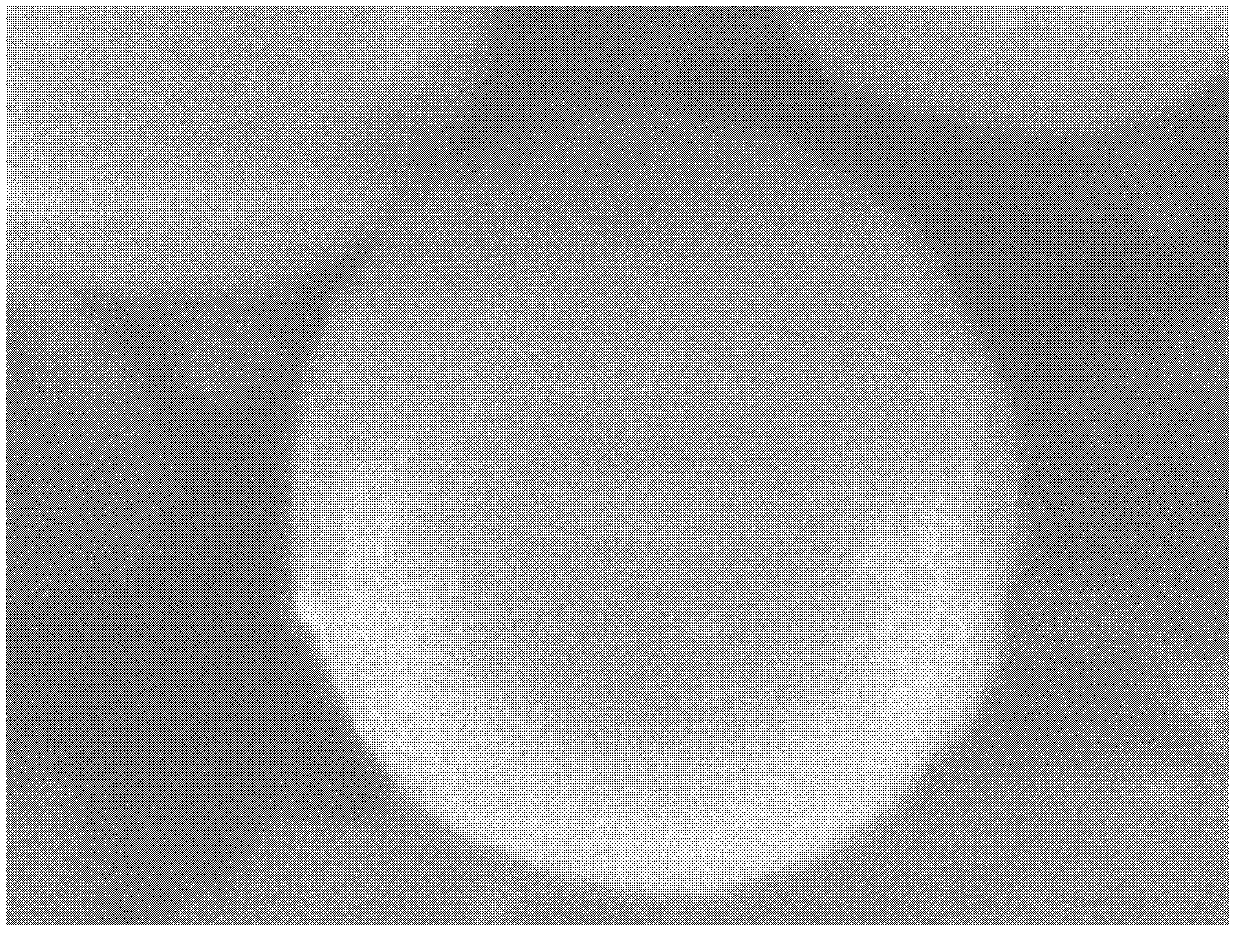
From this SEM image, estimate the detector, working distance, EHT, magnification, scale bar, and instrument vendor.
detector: SEI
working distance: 8 mm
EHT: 5 kV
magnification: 50 K X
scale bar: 100 nm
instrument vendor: JEOL